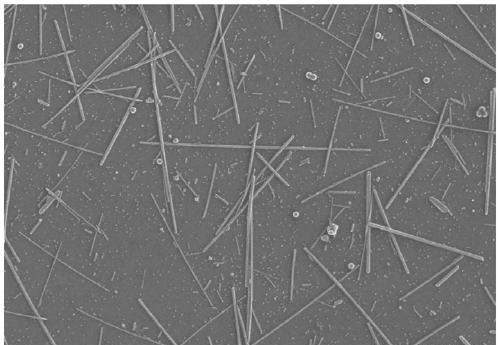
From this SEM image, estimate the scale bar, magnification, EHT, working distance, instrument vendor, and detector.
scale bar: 10000 nm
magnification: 3 K X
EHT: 3 kV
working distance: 9.9 mm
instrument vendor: Hitachi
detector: SE(M)